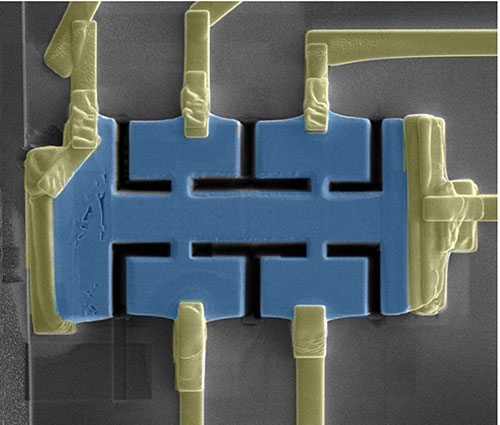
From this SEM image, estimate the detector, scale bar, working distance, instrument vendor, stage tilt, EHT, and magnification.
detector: ETD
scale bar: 10000 nm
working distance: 4.1 mm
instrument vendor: FEI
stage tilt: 0°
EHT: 5 kV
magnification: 3.5 K X